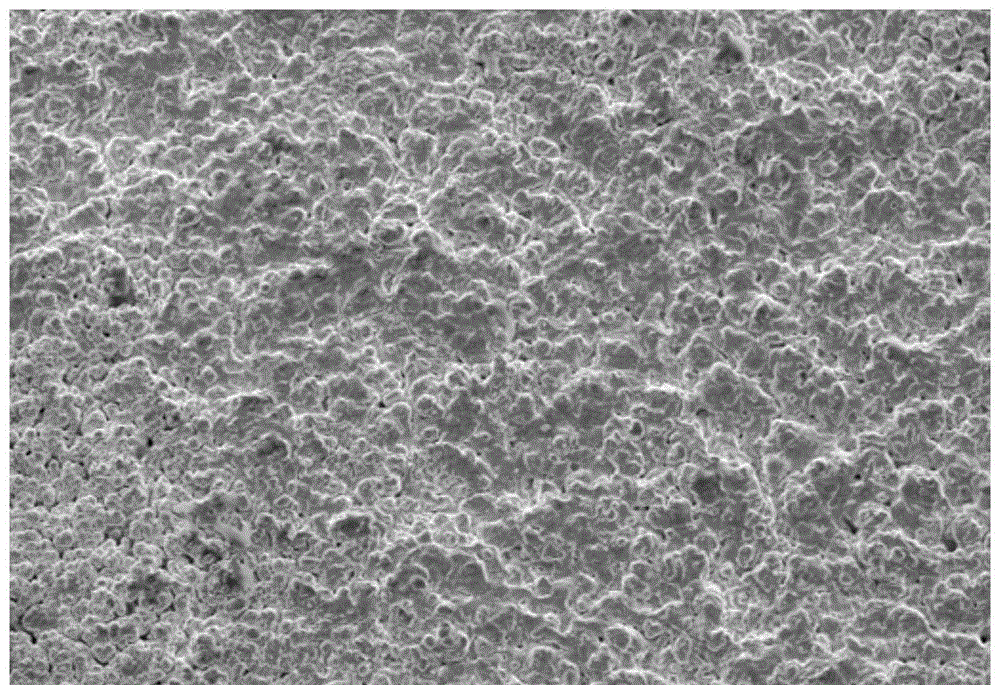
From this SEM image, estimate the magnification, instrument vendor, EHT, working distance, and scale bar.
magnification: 0.5 K X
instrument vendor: Zeiss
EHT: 20 kV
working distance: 10 mm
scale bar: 20000 nm